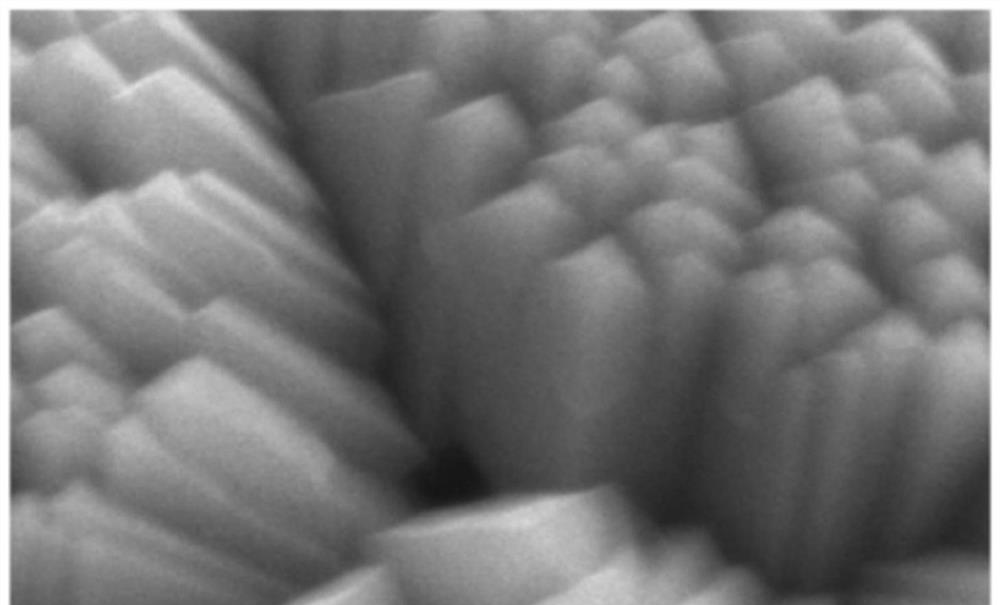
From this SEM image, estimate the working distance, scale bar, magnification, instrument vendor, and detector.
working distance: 8.1 mm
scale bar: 3000 nm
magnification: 15 K X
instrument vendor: JEOL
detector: SE